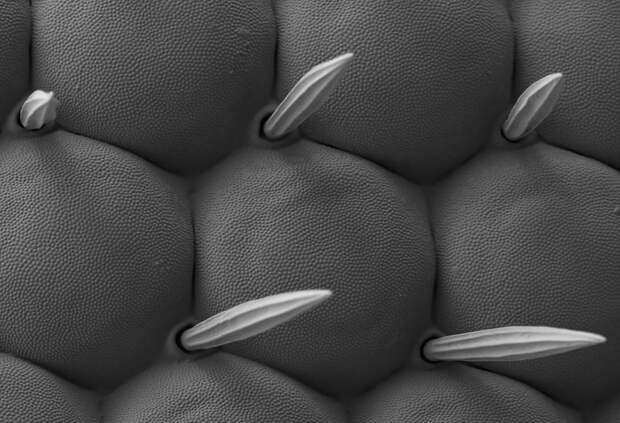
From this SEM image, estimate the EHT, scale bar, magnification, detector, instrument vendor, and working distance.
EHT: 1 kV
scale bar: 2000 nm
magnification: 3 K X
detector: SE2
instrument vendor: Zeiss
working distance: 4.2 mm